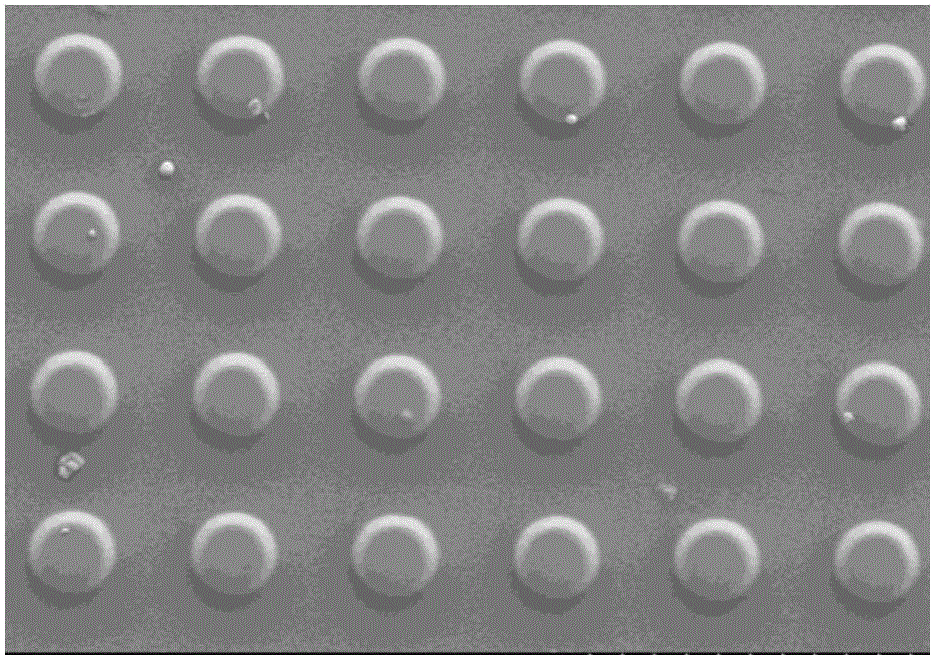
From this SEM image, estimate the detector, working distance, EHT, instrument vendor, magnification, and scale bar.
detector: SE(L)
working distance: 9.3 mm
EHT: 5 kV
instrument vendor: Hitachi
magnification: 2.2 K X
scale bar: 20000 nm